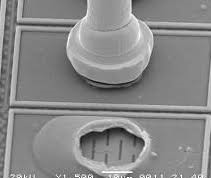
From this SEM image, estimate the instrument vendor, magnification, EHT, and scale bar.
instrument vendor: JEOL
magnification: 1.5 K X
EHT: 20 kV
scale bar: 10000 nm